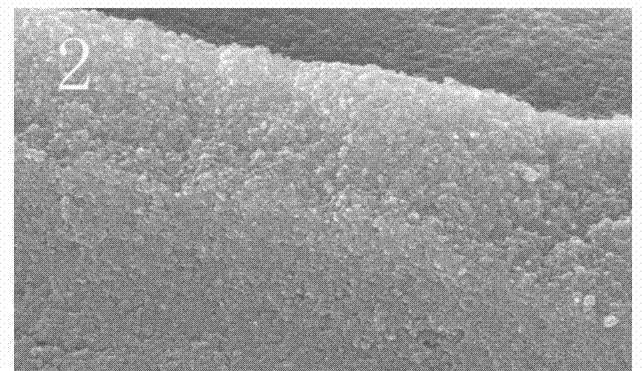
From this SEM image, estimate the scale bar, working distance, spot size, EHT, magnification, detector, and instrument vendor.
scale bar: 1000 nm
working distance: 7.6 mm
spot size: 4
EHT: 25 kV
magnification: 50 K X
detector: SE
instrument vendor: FEI